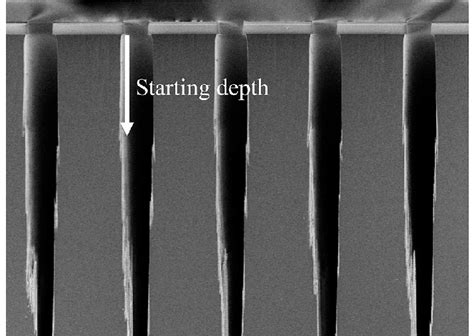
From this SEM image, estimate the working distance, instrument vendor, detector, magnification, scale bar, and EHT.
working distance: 0.2 mm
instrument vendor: Hitachi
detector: SE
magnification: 1.3 K X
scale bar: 40000 nm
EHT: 1.5 kV